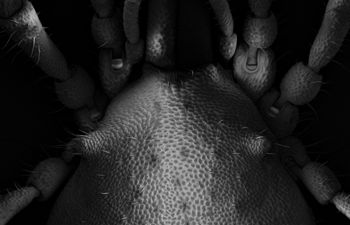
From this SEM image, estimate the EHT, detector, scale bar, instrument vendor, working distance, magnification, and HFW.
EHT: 5 kV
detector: InLens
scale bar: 100000 nm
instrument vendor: Zeiss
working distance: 12.2 mm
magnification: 0.217 K X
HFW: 1397 µm